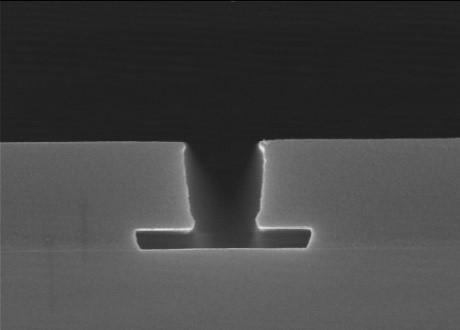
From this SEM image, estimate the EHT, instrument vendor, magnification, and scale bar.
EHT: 15 kV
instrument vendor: Hitachi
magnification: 30 K X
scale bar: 1000 nm